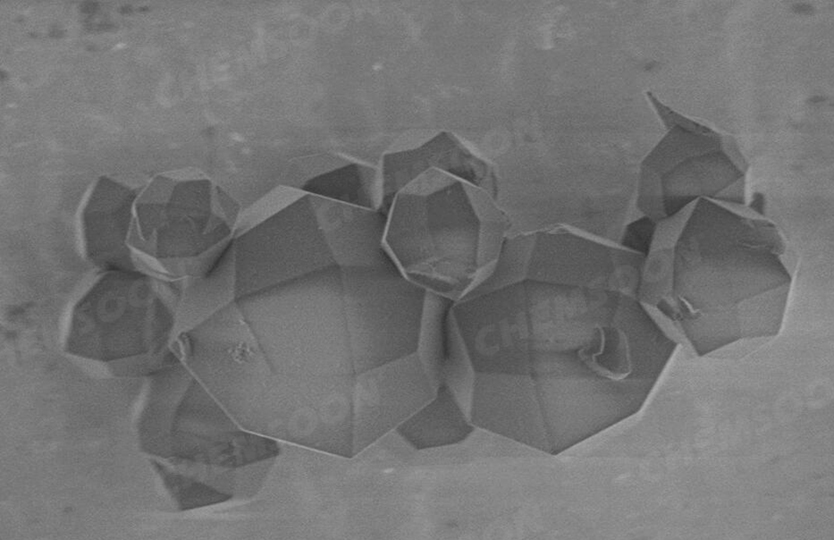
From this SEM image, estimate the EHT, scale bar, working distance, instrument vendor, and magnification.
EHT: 1 kV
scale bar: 10000 nm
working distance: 3.8 mm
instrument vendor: Hitachi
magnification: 4.5 K X